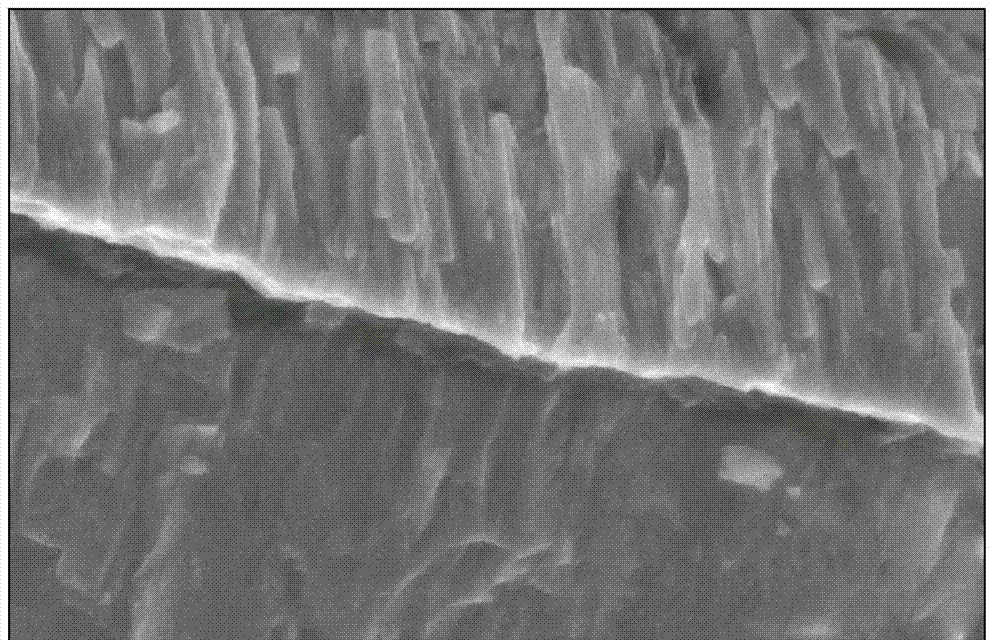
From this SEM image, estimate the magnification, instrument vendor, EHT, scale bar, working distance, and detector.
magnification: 30 K X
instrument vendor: JEOL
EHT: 5 kV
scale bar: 100 nm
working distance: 7.8 mm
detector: SEI